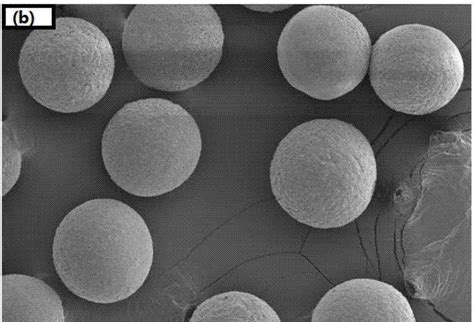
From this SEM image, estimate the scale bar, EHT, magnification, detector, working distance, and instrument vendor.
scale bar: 100000 nm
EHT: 5 kV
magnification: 0.32 K X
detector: SE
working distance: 9.2 mm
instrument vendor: Hitachi